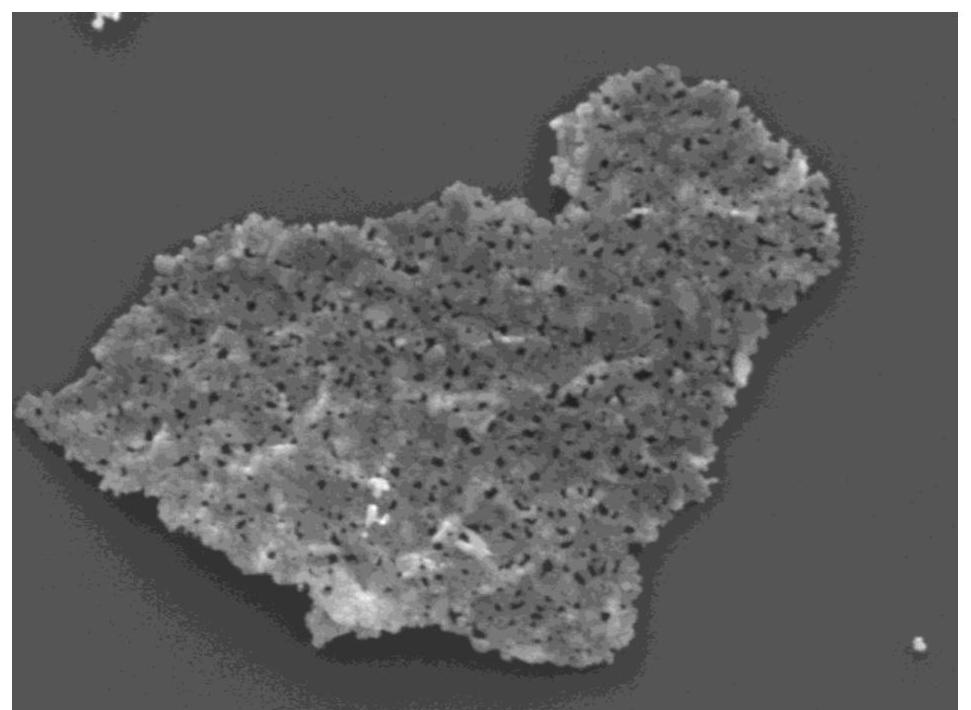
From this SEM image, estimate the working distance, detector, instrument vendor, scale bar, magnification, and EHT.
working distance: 8.4 mm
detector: LED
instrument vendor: JEOL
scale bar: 1000 nm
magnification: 25 K X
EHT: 3 kV